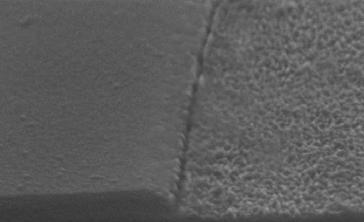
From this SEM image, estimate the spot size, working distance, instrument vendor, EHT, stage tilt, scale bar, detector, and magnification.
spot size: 2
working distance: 7.9 mm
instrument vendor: FEI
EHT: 12 kV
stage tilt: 0°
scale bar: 500 nm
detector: SE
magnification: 54.314 K X